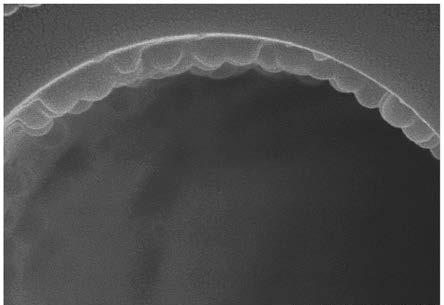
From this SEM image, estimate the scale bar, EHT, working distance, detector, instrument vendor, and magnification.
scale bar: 500 nm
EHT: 10 kV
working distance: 9.7 mm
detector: SE(U)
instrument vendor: Hitachi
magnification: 80 K X